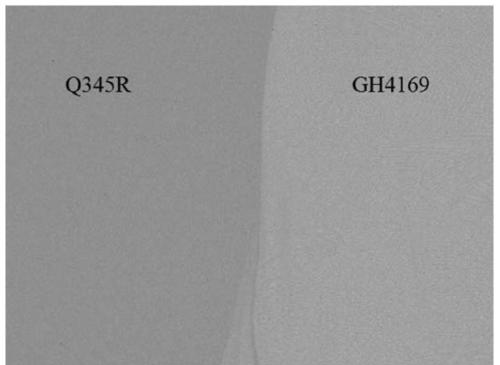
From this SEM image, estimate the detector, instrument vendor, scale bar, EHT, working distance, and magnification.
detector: BED-C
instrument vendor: JEOL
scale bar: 100000 nm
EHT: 20 kV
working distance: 10 mm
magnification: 0.1 K X